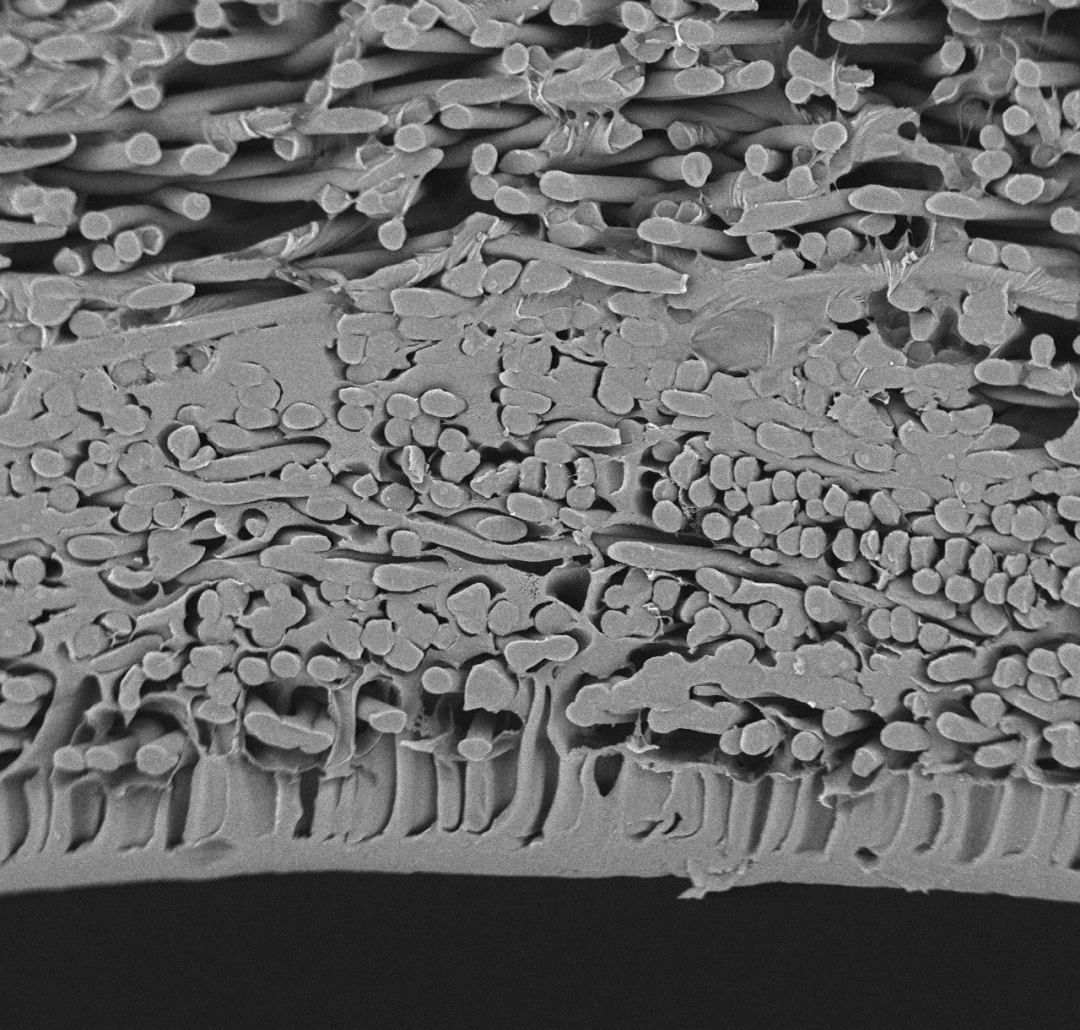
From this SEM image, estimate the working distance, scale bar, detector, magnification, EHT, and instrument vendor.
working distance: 5.6 mm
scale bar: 50000 nm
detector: BSE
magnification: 0.6 K X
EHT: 15 kV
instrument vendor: other